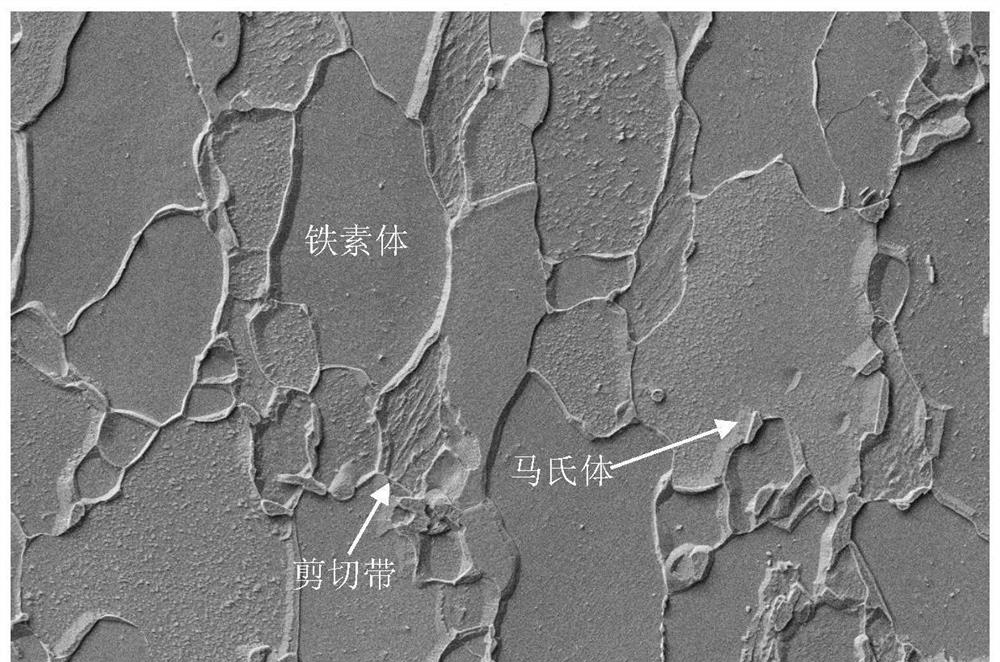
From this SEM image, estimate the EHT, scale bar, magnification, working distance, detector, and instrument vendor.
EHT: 5 kV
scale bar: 2000 nm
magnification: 5 K X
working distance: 9.2 mm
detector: SE2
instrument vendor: Zeiss